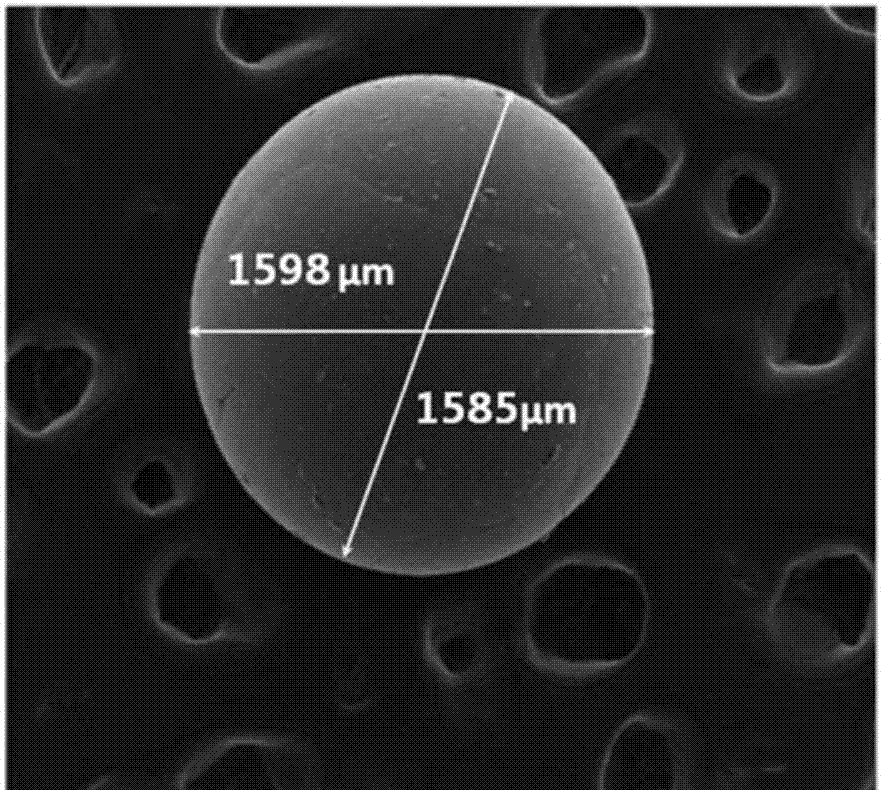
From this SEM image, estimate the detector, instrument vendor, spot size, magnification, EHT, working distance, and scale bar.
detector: ETD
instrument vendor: FEI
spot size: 3.5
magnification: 0.08 K X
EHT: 20 kV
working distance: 12.8 mm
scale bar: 500000 nm